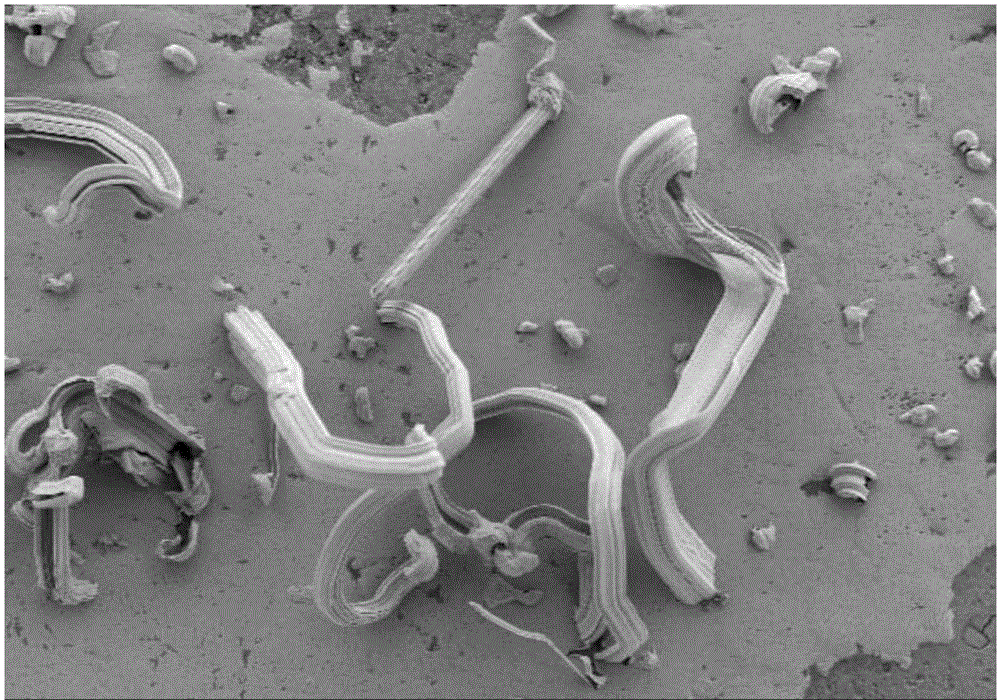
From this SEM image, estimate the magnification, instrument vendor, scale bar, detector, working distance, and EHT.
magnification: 1 K X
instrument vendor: Hitachi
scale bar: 50000 nm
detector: SE(L)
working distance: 13.5 mm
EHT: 5 kV